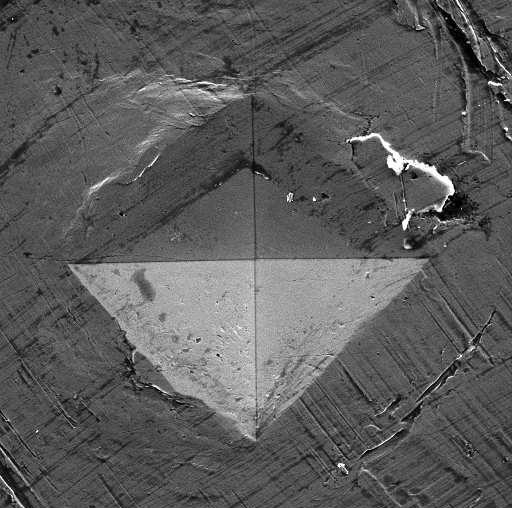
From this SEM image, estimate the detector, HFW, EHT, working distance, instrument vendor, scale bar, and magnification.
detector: SE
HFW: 180.1 µm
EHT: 15 kV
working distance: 13.81 mm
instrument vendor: Tescan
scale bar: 50000 nm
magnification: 1.35 K X